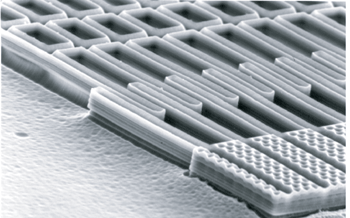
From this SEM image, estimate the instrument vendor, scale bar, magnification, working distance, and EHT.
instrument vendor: JEOL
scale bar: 10000 nm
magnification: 2 K X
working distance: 39 mm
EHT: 15 kV